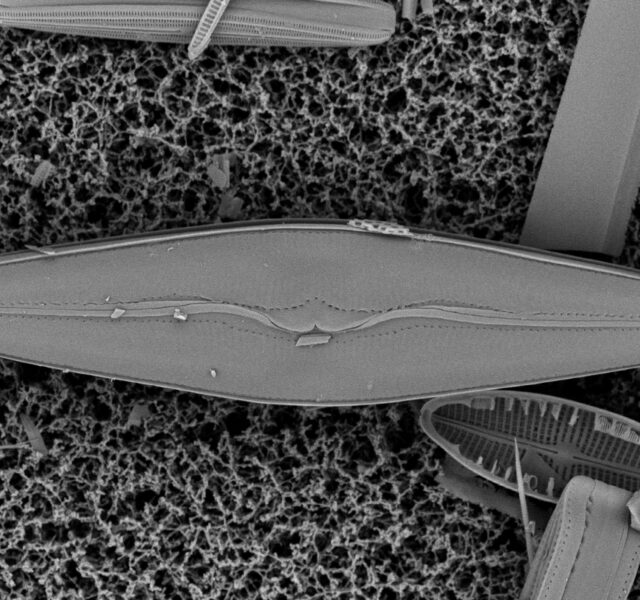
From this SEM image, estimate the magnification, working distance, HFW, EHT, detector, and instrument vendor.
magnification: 6.2 K X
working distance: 4.998 mm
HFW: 83.7 µm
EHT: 10 kV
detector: BSD Full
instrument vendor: Thermo